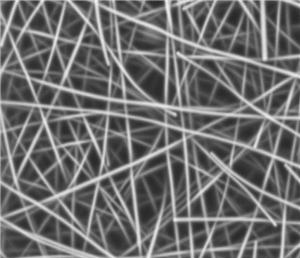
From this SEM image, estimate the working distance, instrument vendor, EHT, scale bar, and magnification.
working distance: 5.1 mm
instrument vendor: Zeiss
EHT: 3 kV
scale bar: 200 nm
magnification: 30 K X